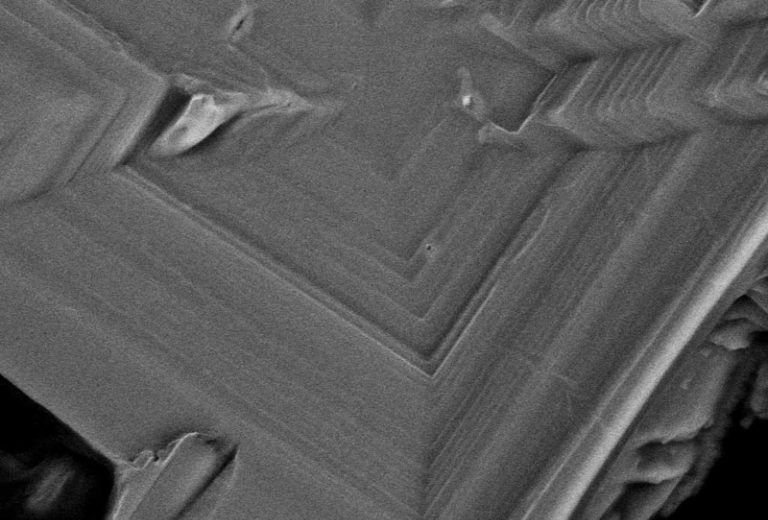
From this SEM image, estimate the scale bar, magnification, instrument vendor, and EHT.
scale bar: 10000 nm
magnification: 2.5 K X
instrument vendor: JEOL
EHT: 15 kV